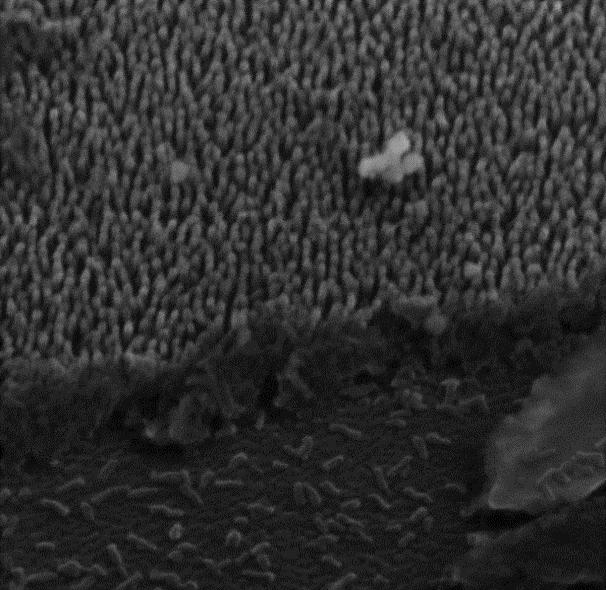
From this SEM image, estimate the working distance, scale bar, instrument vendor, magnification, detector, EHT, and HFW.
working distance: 5.275 mm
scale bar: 200 nm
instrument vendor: Tescan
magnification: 161.75 K X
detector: InBeam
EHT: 15 kV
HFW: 1.34 µm